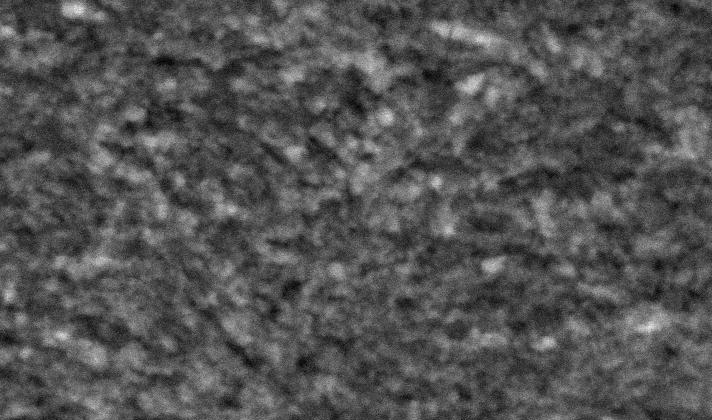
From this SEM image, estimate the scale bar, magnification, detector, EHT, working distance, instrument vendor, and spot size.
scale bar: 5000 nm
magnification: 5 K X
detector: SE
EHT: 20 kV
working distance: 8.3 mm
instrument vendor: FEI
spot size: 3